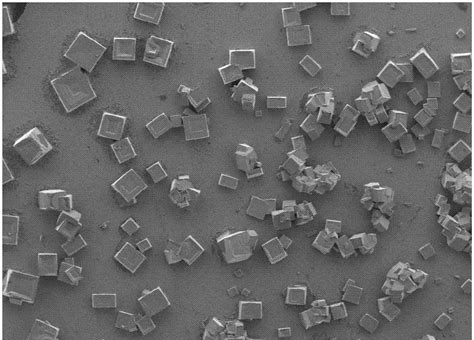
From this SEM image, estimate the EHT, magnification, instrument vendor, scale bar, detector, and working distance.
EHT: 3 kV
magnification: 0.1 K X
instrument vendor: JEOL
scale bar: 100000 nm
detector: LED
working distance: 8.1 mm